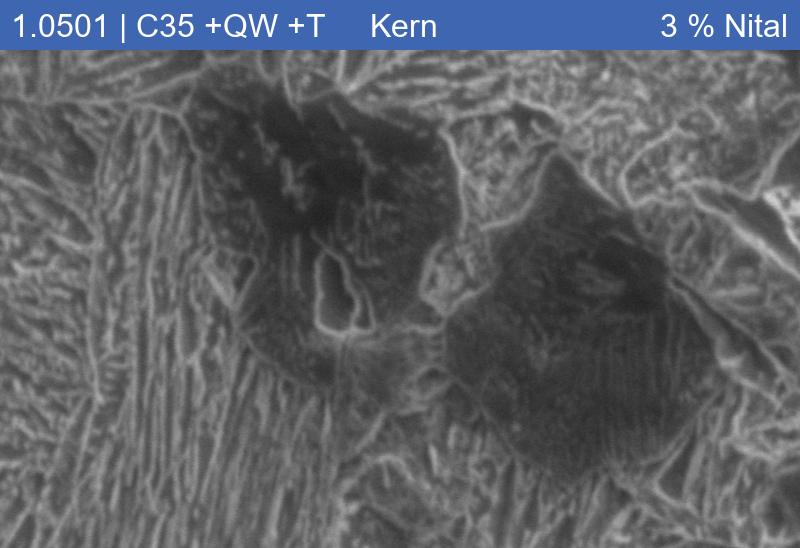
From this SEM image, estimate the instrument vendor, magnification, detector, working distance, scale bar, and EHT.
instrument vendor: Zeiss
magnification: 10 K X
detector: SE1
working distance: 9.66 mm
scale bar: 1000 nm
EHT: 5 kV